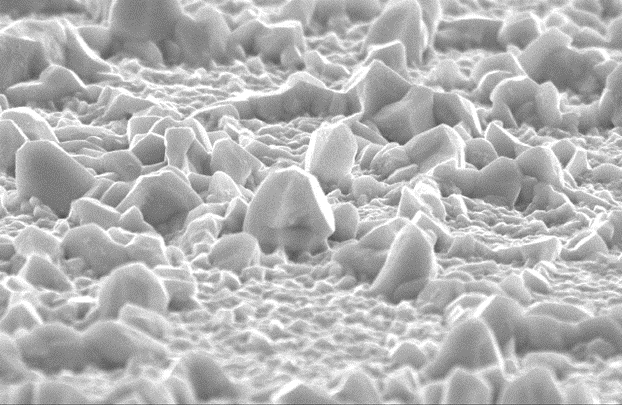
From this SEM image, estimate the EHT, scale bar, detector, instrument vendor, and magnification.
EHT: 20 kV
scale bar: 5000 nm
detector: SEI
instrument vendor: JEOL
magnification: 5 K X